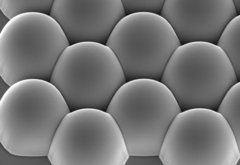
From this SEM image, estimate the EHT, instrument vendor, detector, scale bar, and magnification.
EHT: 15 kV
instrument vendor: Hitachi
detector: SE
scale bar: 30000 nm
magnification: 1.5 K X